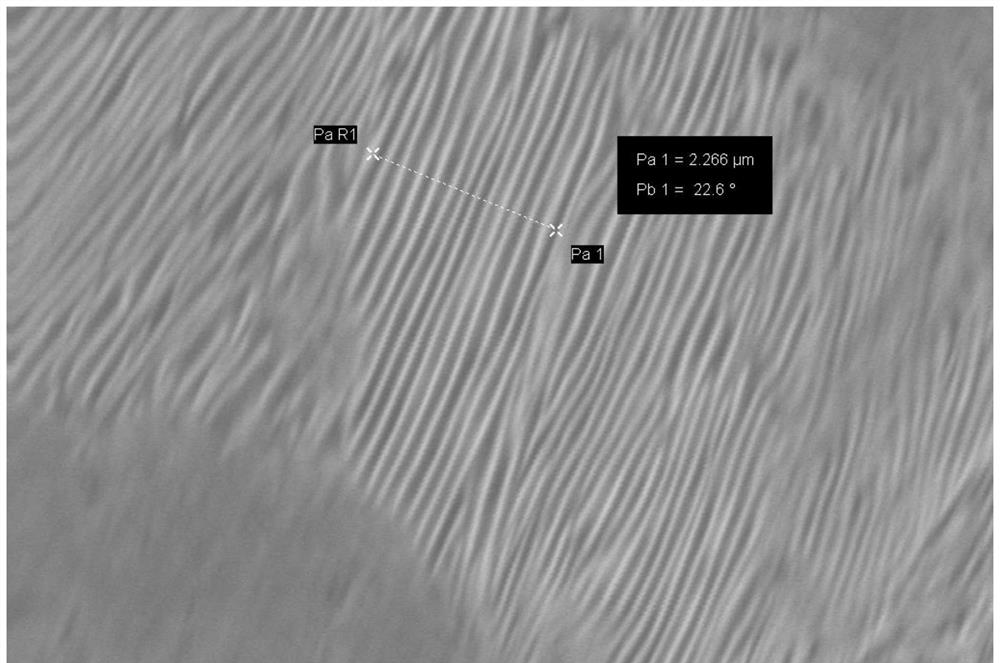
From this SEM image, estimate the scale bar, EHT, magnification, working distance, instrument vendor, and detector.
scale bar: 1000 nm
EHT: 20 kV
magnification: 10.14 K X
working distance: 10.5 mm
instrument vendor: Zeiss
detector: SE1